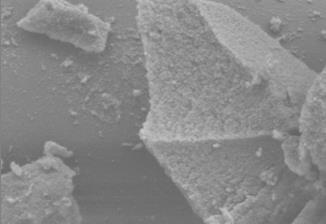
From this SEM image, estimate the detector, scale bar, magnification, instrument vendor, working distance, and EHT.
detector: SE(UL)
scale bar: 2000 nm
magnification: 20 K X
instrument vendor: Hitachi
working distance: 14 mm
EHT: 3 kV